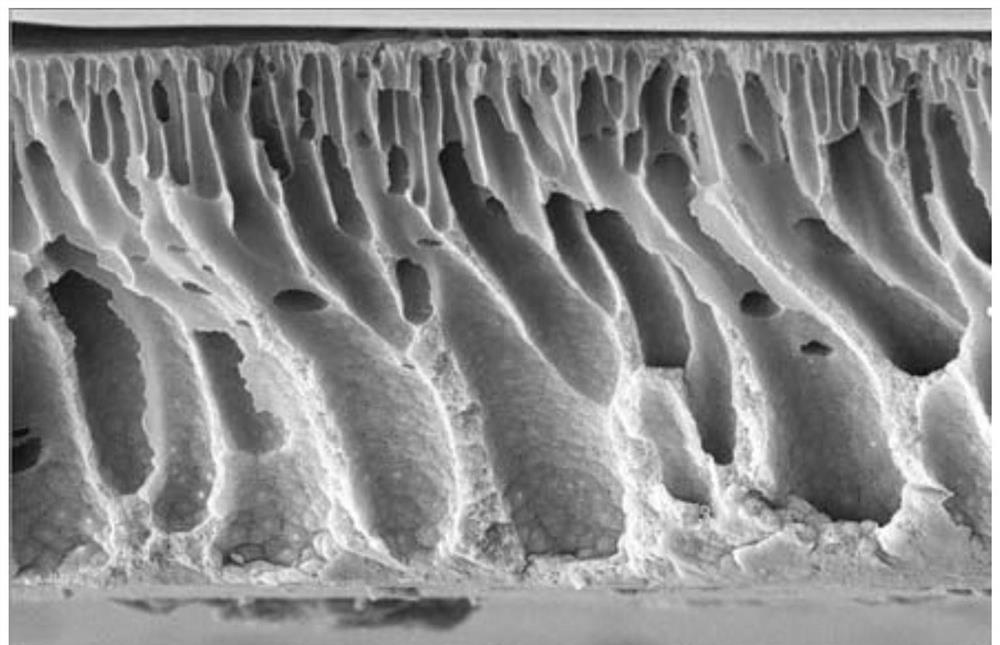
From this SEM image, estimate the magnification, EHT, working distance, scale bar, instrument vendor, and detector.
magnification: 0.5 K X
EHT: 15 kV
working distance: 8.2 mm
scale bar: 100000 nm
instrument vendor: FEI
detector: ETD